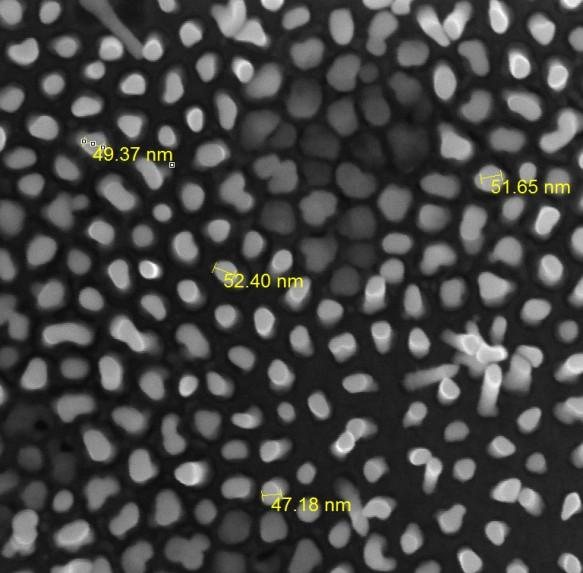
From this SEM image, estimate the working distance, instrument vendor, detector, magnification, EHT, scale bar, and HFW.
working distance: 2.651 mm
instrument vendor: Tescan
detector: InBeam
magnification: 150 K X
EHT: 15 kV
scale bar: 200 nm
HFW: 1.445 µm